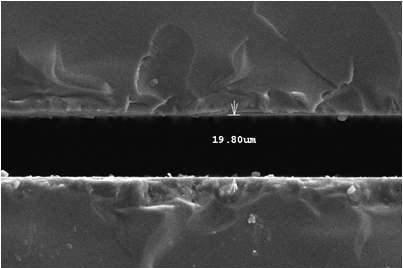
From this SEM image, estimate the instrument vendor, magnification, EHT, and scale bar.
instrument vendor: JEOL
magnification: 1 K X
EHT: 15 kV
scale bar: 50000 nm